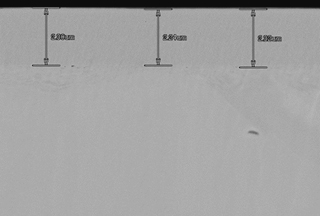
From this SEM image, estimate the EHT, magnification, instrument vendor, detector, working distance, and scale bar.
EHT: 10 kV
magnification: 10 K X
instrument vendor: Hitachi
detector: LA50(UL)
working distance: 8.5 mm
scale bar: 5000 nm